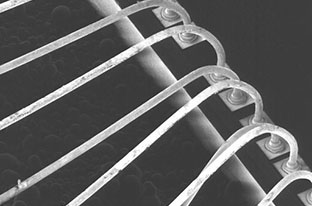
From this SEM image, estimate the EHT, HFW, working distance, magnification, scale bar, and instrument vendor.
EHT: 30 kV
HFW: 762 µm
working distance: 17 mm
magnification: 0.336 K X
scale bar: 200000 nm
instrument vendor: FEI